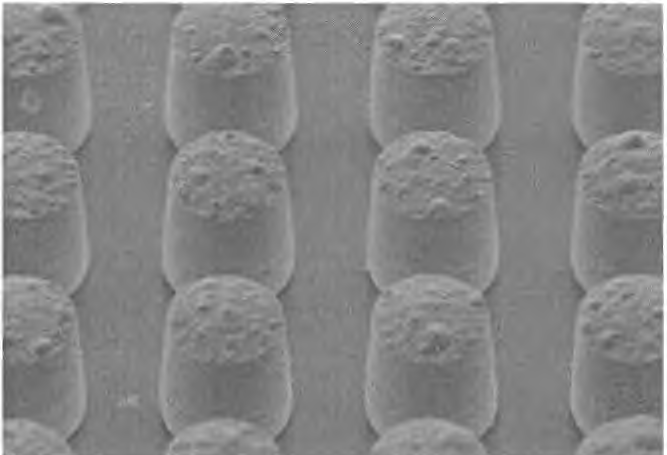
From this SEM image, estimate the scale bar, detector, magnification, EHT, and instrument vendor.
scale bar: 50000 nm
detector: SED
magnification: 0.7 K X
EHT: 20 kV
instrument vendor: JEOL